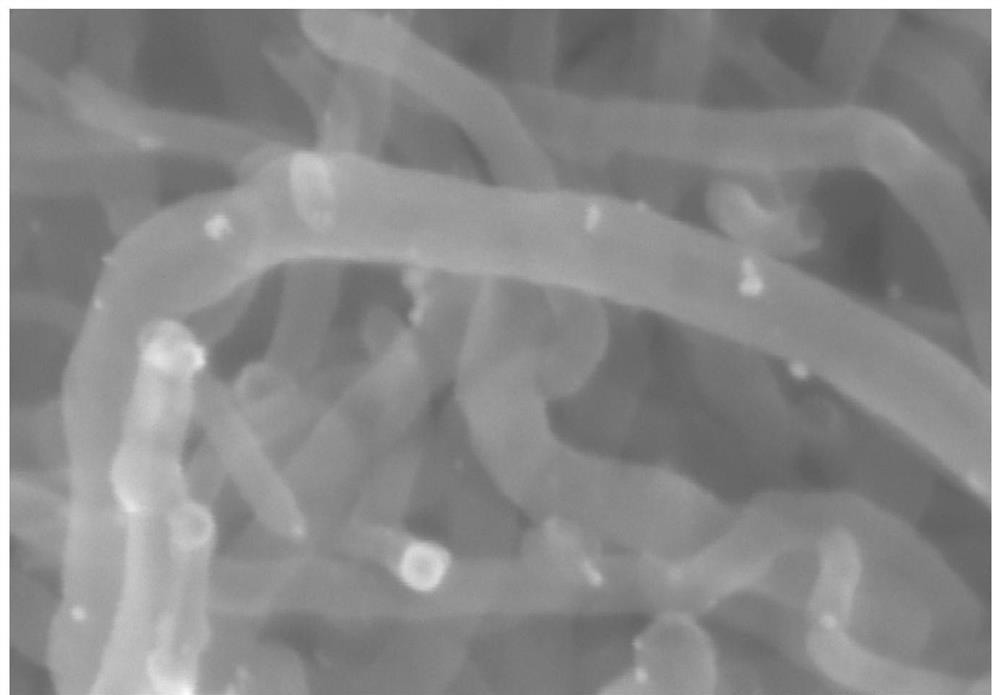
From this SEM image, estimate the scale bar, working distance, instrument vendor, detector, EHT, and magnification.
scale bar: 100 nm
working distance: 11.4 mm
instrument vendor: Hitachi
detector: SE(UL)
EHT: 10 kV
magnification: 300 K X